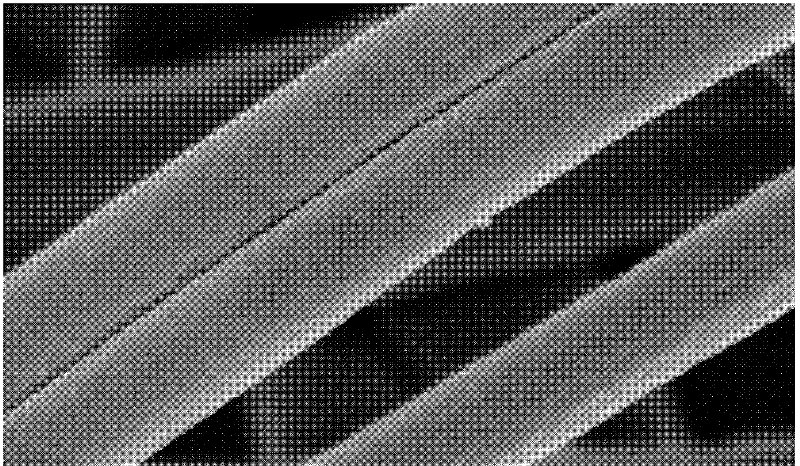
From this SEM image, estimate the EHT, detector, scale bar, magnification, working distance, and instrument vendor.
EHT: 3 kV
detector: SE(M)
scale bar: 1000 nm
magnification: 50 K X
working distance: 8.7 mm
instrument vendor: Hitachi